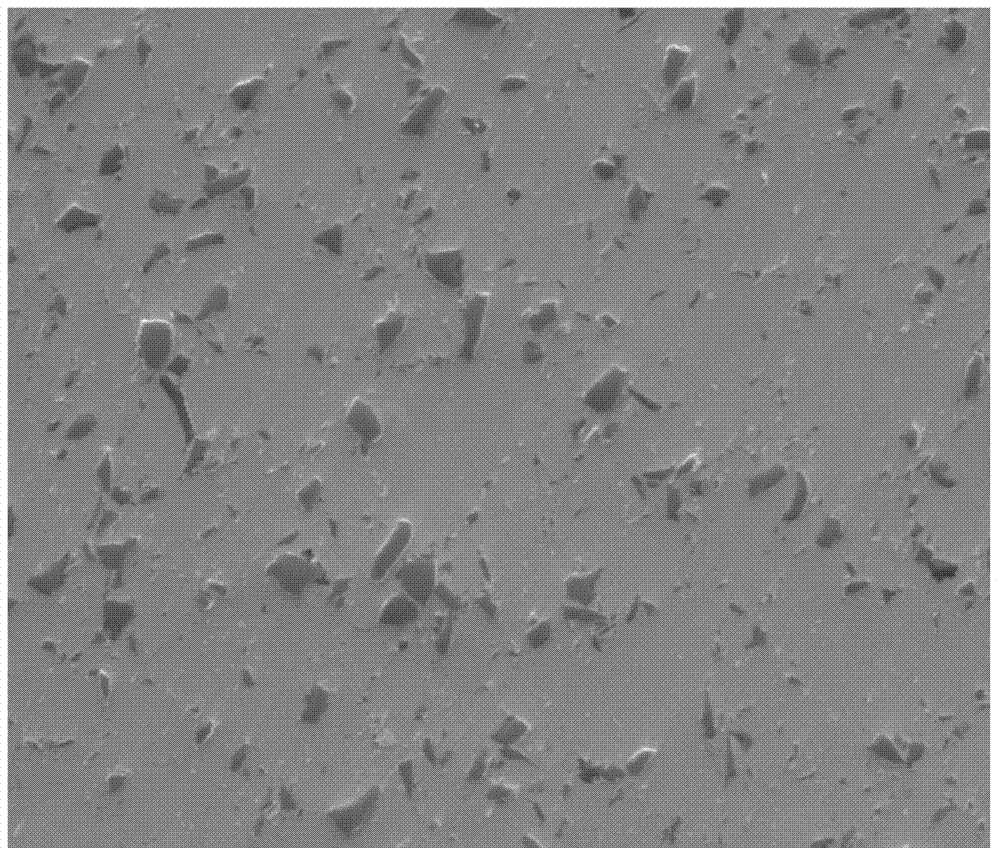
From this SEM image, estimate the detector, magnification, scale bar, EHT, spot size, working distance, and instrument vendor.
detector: ETD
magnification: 2 K X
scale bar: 50000 nm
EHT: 10 kV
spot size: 3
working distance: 10 mm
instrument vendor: FEI